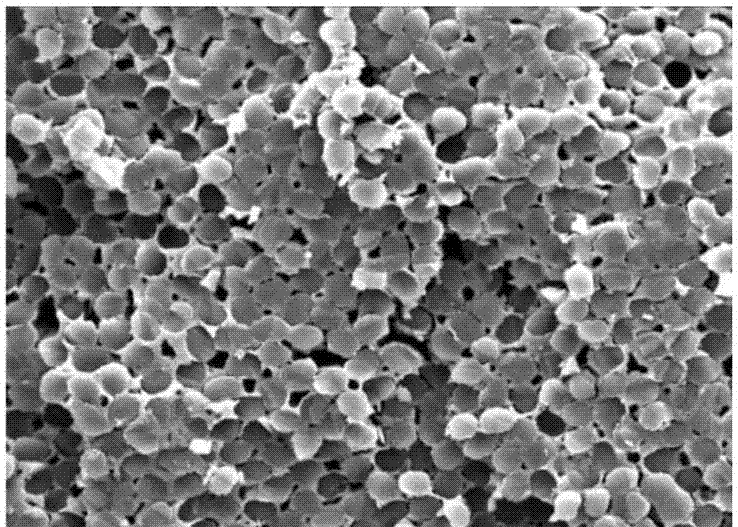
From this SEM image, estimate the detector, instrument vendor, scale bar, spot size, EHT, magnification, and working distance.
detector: ETD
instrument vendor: FEI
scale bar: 50000 nm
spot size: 4.5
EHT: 20 kV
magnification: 2 K X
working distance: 10.7 mm